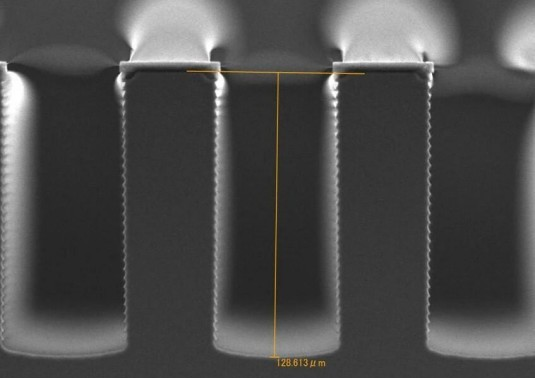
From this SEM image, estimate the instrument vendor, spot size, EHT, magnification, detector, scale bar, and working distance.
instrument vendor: JEOL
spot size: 37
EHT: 15 kV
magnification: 0.5 K X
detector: SEI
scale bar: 50000 nm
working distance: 20 mm